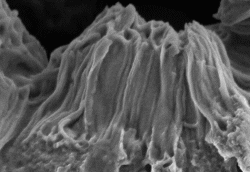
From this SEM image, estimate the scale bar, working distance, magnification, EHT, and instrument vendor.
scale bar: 2000 nm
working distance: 20.2 mm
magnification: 20 K X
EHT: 3 kV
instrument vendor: Hitachi